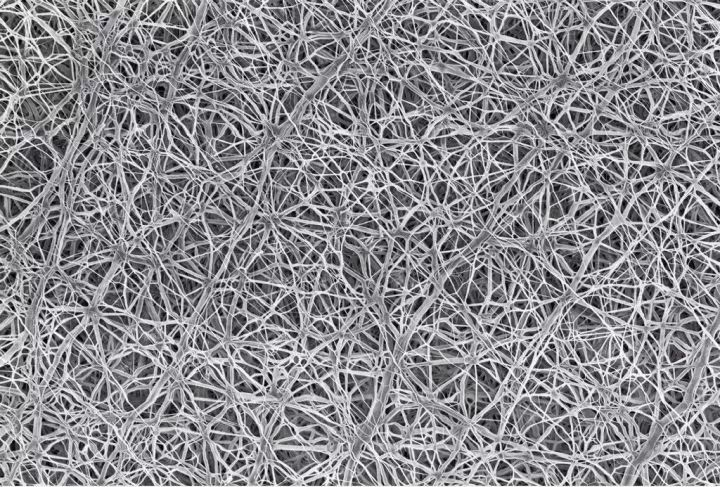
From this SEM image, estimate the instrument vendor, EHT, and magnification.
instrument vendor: Zeiss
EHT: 2 kV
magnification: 5 K X